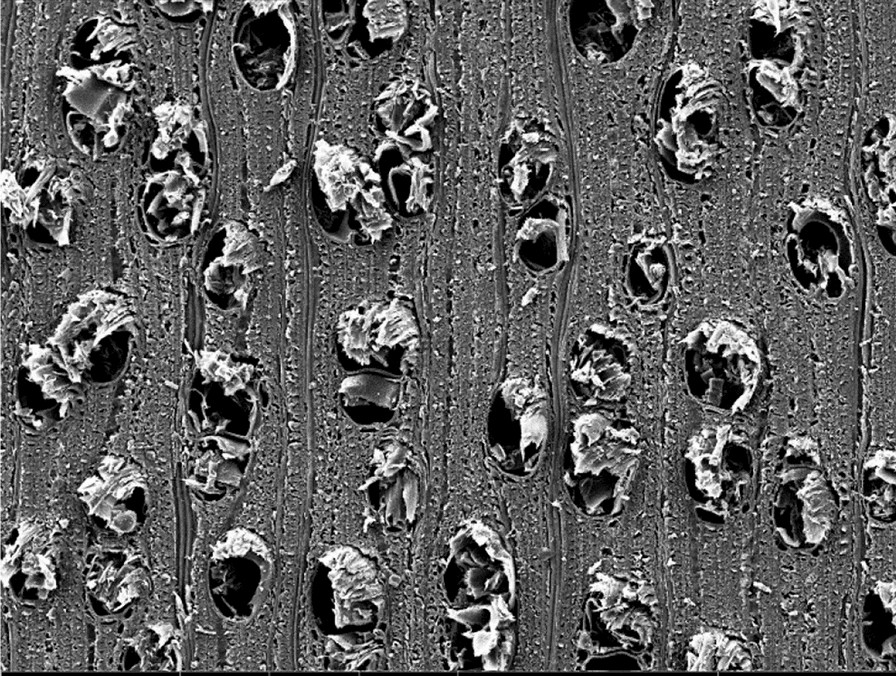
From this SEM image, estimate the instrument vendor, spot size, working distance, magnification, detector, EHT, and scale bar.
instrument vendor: other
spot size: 2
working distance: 15.78 mm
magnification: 0.2 K X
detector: SEI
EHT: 20 kV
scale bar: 60000 nm